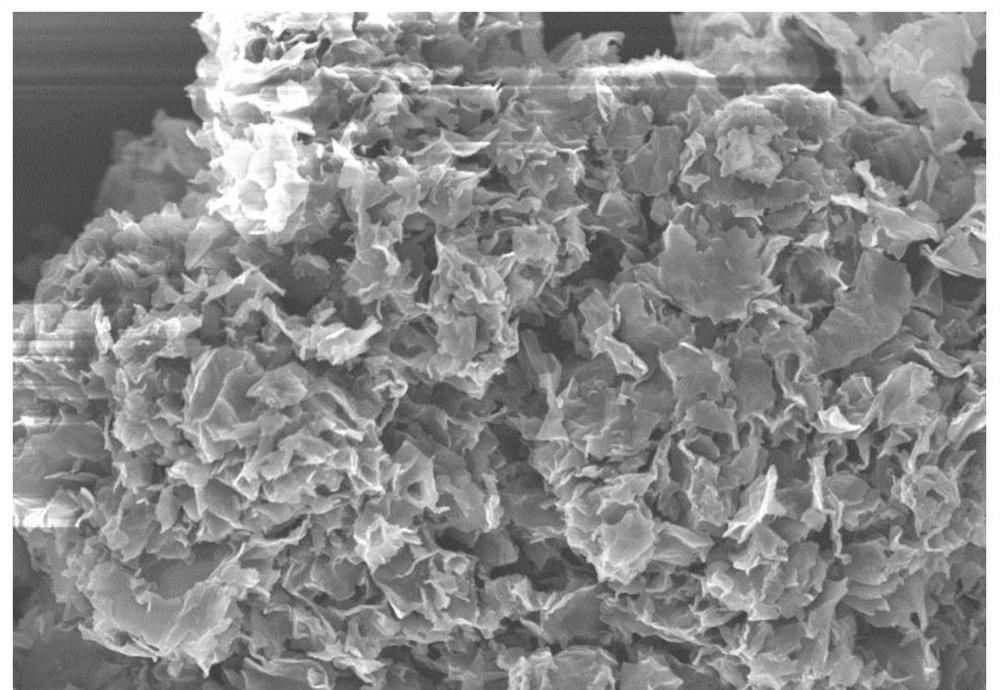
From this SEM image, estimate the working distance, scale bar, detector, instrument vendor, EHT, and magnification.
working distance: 7.2 mm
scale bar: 20000 nm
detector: SE(U)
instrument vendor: Hitachi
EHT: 10 kV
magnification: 2 K X